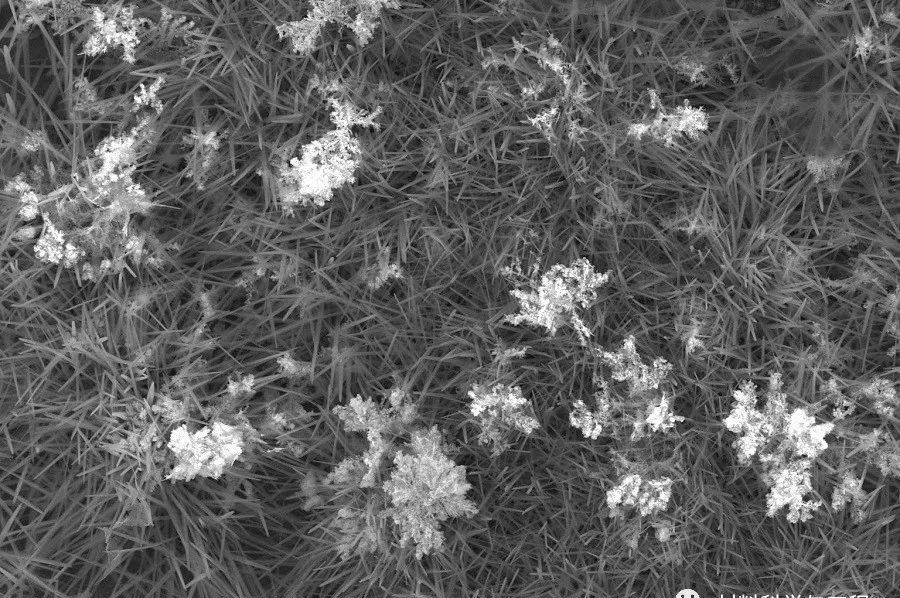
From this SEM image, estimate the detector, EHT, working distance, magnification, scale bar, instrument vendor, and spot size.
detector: SE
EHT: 20 kV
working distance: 10.1 mm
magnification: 10 K X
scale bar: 10000 nm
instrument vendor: FEI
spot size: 3